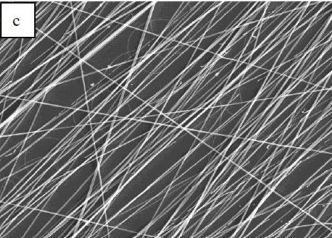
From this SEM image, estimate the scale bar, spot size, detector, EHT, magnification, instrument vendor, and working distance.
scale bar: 50000 nm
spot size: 3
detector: ETD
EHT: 20 kV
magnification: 1 K X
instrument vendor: FEI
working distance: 11.3 mm